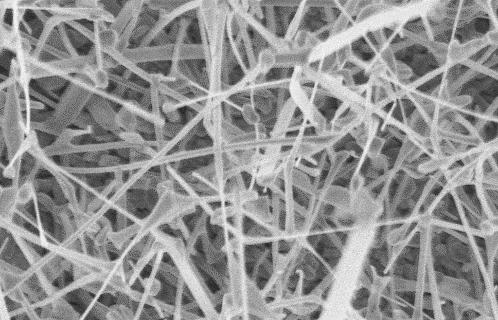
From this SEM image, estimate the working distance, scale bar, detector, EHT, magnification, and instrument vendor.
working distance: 10.6 mm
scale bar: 200 nm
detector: InLens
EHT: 3 kV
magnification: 50 K X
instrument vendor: Zeiss